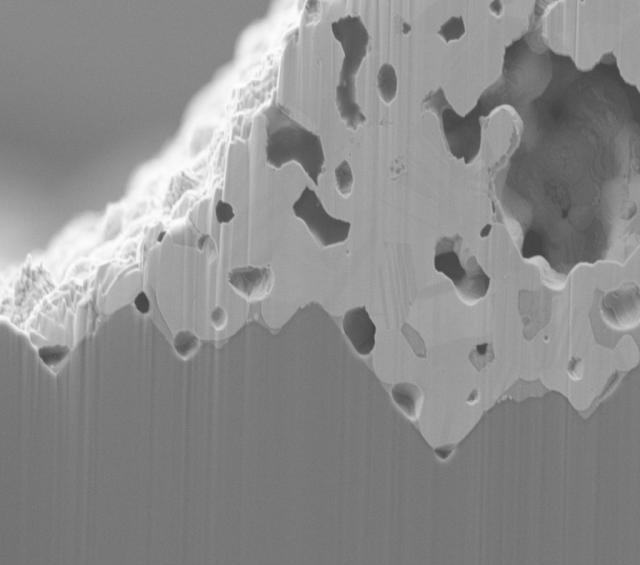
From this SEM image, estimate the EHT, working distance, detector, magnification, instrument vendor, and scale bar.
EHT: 4 kV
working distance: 3.9 mm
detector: SE2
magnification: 5 K X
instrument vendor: Zeiss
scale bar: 1000 nm